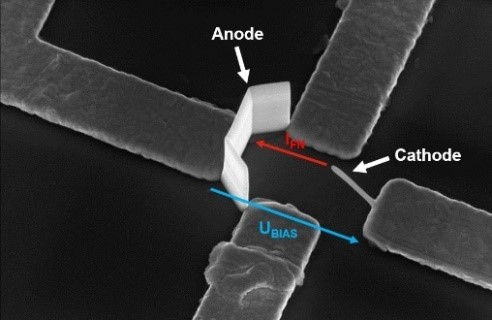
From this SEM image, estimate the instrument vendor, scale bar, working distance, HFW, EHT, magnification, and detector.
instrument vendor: FEI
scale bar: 1000 nm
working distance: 4.1 mm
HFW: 6.34 µm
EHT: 5 kV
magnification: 20.044 K X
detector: TLD